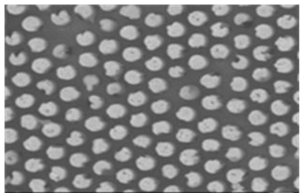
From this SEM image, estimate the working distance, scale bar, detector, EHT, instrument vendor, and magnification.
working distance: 7.5 mm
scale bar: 1000 nm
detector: SEI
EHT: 10 kV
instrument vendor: JEOL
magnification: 10 K X